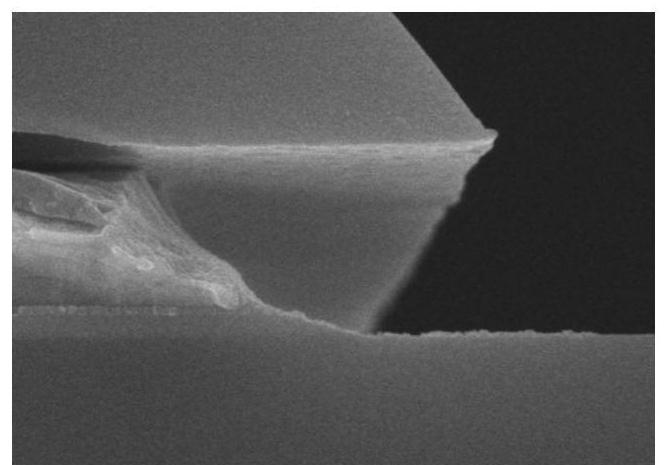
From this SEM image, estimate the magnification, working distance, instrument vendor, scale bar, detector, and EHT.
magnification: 50 K X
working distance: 12 mm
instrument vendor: Hitachi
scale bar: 1000 nm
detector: SE(U)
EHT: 10 kV